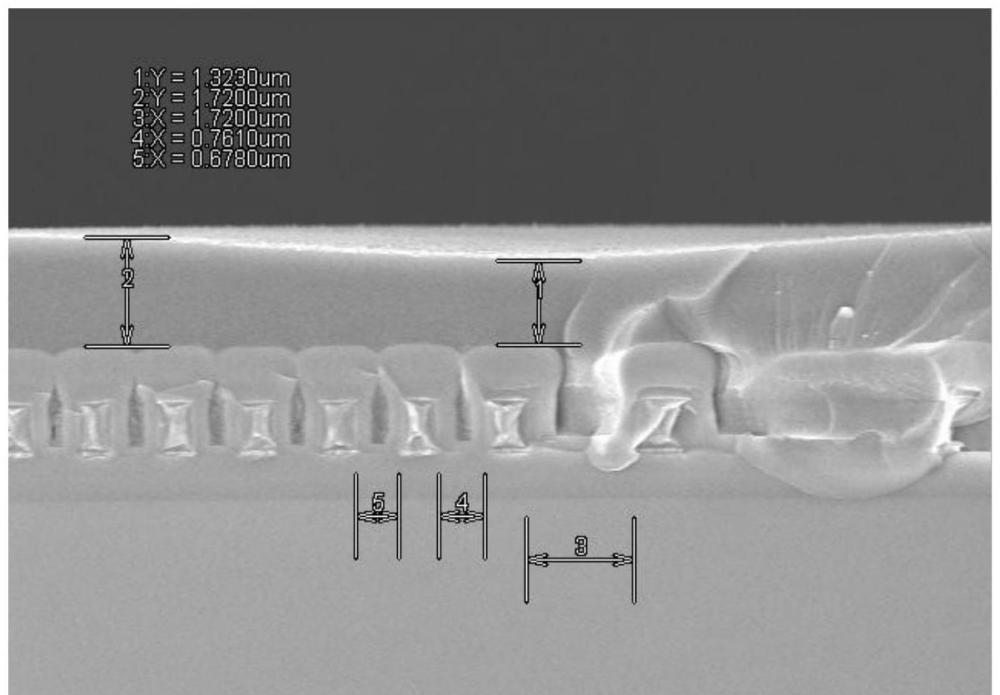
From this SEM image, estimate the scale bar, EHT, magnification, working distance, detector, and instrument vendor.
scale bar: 5000 nm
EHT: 5 kV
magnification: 8 K X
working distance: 14.4 mm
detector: SE(M)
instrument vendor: Hitachi